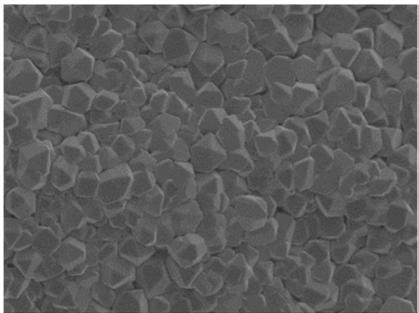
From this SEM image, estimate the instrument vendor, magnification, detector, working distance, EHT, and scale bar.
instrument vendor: Hitachi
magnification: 5 K X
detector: SE(M)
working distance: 9.3 mm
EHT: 3 kV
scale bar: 10000 nm